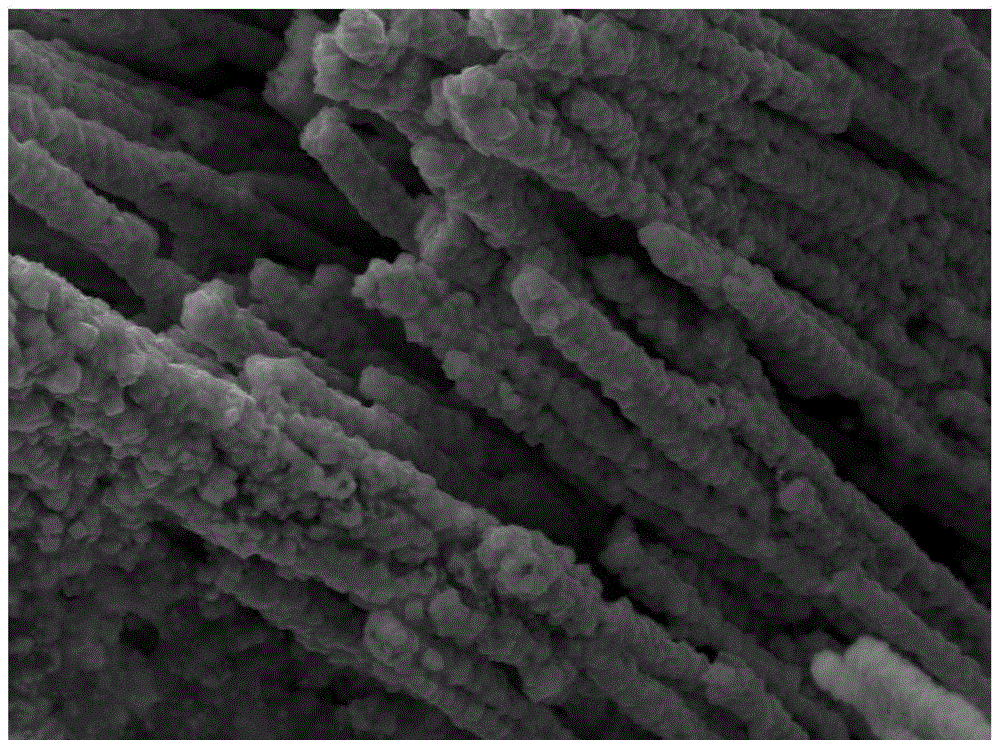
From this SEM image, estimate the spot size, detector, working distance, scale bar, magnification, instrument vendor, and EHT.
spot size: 3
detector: SE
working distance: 4.6 mm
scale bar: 2000 nm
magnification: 10 K X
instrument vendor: FEI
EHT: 10 kV